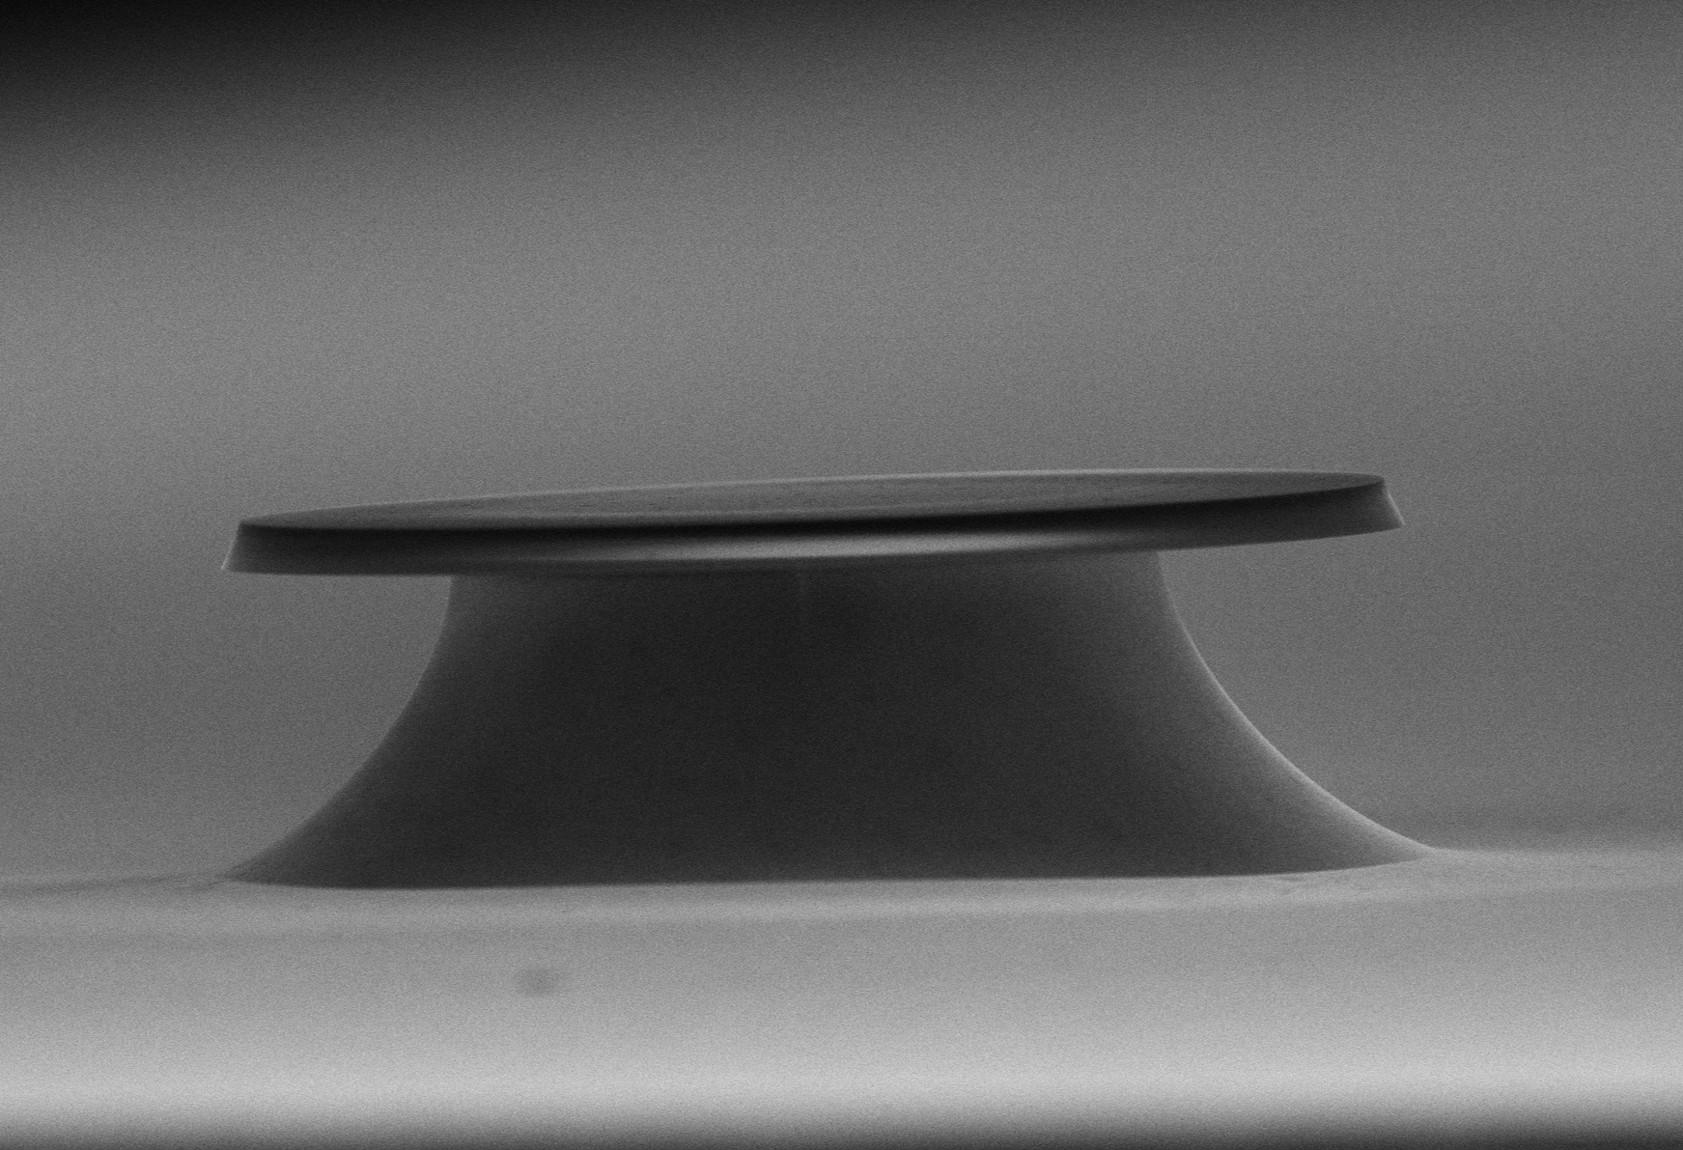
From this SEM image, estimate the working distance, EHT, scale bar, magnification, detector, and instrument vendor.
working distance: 10.5 mm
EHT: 10 kV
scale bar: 30000 nm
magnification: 1.8 K X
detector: SE(U)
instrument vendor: Hitachi